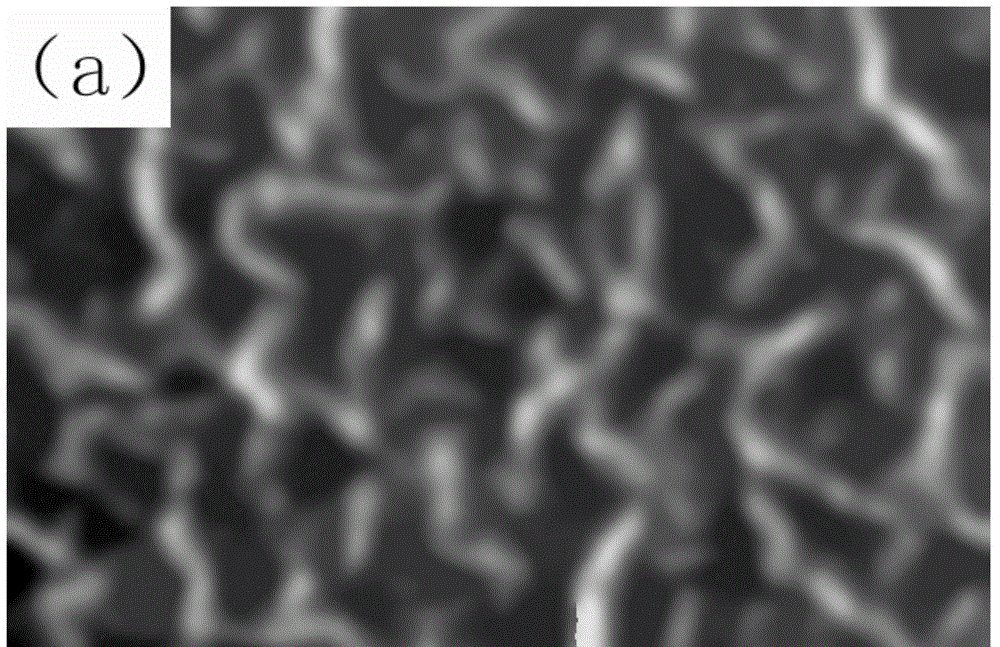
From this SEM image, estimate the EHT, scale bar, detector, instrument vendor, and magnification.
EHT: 20 kV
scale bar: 500 nm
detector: SEI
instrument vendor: JEOL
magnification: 50 K X